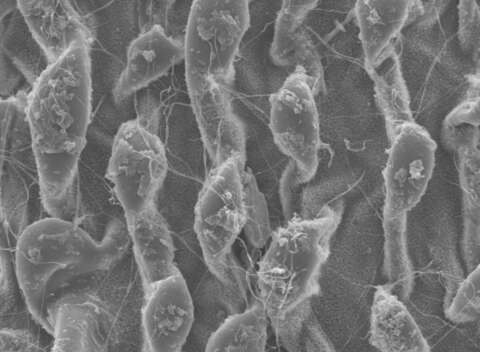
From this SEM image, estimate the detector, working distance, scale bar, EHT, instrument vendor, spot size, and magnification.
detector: SE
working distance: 16.1 mm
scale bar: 10000 nm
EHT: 10 kV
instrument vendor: FEI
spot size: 3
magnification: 1.6 K X